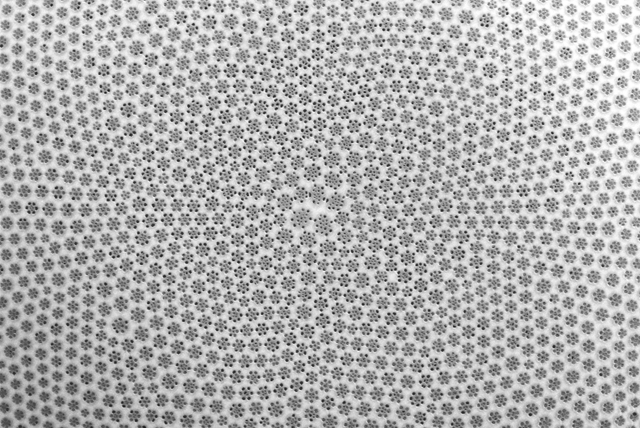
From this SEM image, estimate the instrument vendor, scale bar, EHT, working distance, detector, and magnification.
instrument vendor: Zeiss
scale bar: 10000 nm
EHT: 20 kV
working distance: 11.7 mm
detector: SE2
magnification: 4.22 K X